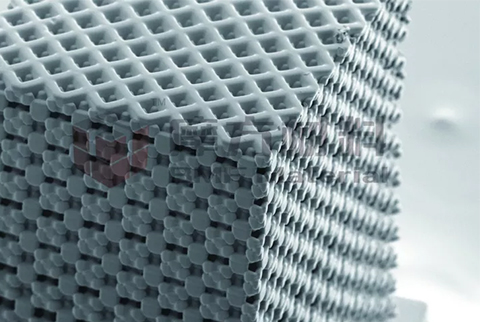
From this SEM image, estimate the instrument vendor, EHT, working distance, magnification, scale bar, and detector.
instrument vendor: Zeiss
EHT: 5 kV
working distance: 7.2 mm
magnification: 0.105 K X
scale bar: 100000 nm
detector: SE2